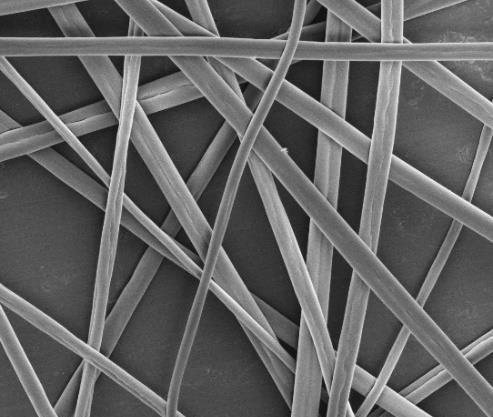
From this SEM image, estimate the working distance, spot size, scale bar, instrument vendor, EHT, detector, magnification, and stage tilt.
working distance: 12 mm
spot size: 3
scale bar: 10000 nm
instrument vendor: FEI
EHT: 5 kV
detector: ETD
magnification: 5 K X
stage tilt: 0°